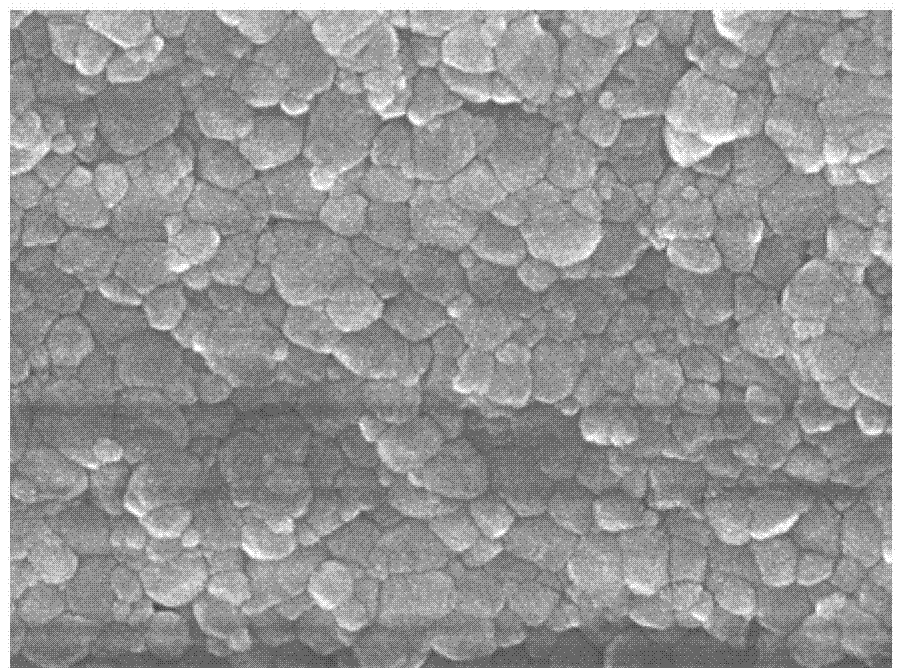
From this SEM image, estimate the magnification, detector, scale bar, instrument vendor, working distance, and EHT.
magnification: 50 K X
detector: SEI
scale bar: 100 nm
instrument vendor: JEOL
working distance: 7.7 mm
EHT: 10 kV